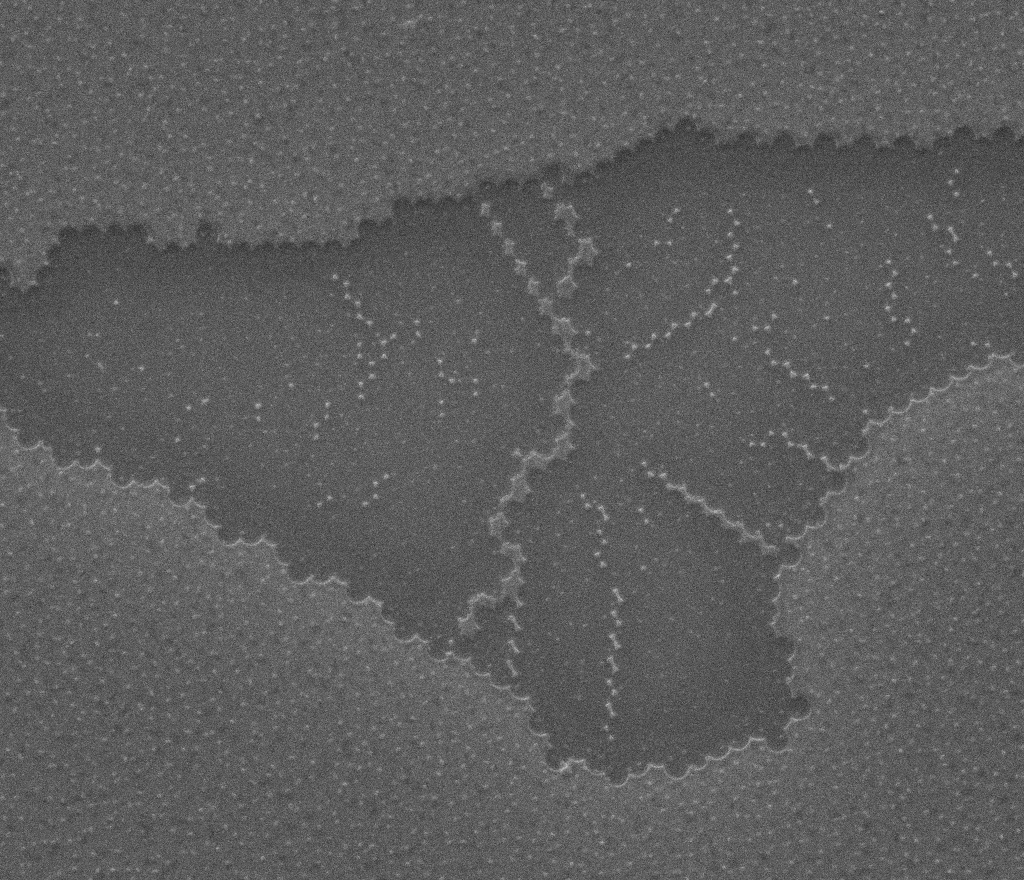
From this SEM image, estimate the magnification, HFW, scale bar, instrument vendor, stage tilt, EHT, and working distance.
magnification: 12.186 K X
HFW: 22.2 µm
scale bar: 5000 nm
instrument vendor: FEI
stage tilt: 0°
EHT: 25 kV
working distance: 12 mm